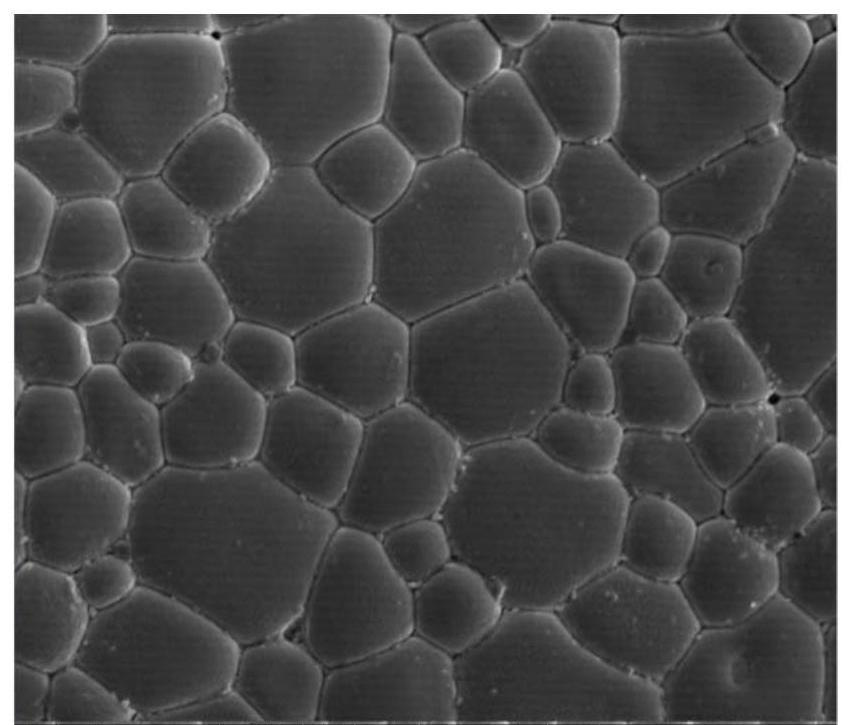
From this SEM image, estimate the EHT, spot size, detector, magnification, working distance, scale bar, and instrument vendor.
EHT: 10 kV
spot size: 3.5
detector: ETD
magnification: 5 K X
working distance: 5 mm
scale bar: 10000 nm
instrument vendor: FEI